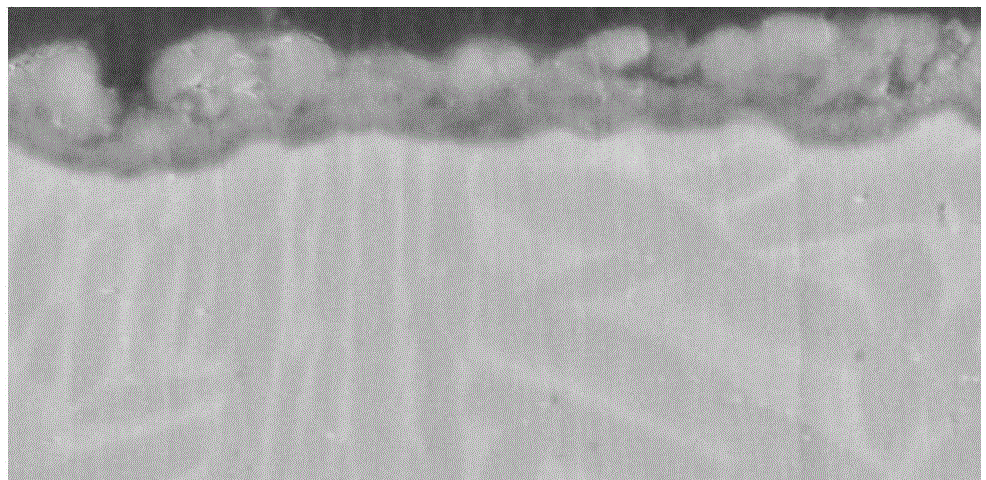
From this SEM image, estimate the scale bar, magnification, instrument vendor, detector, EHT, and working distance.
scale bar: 10000 nm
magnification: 5 K X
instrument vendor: Hitachi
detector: SE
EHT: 20 kV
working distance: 11.4 mm